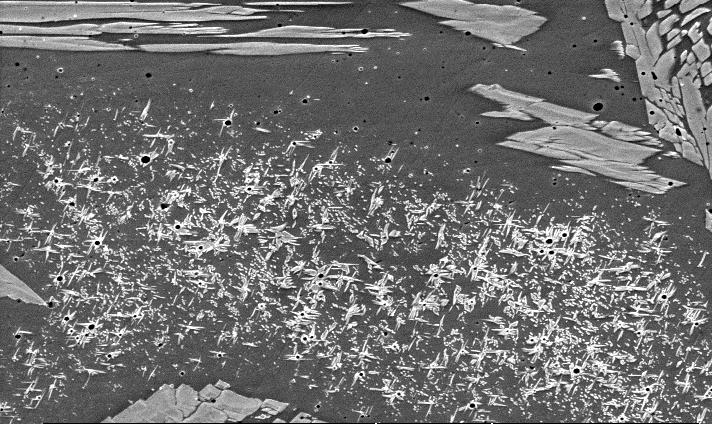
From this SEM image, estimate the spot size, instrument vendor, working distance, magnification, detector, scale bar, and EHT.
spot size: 5.5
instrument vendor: FEI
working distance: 10 mm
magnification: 2 K X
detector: SE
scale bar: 20000 nm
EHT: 20 kV